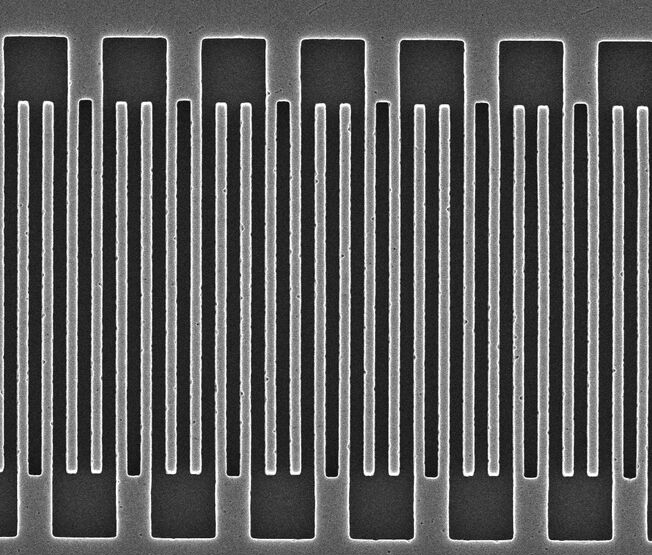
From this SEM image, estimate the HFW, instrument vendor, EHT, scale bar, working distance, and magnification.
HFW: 10.5 µm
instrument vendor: FEI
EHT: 15 kV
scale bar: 5000 nm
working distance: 5.2 mm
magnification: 12.142 K X